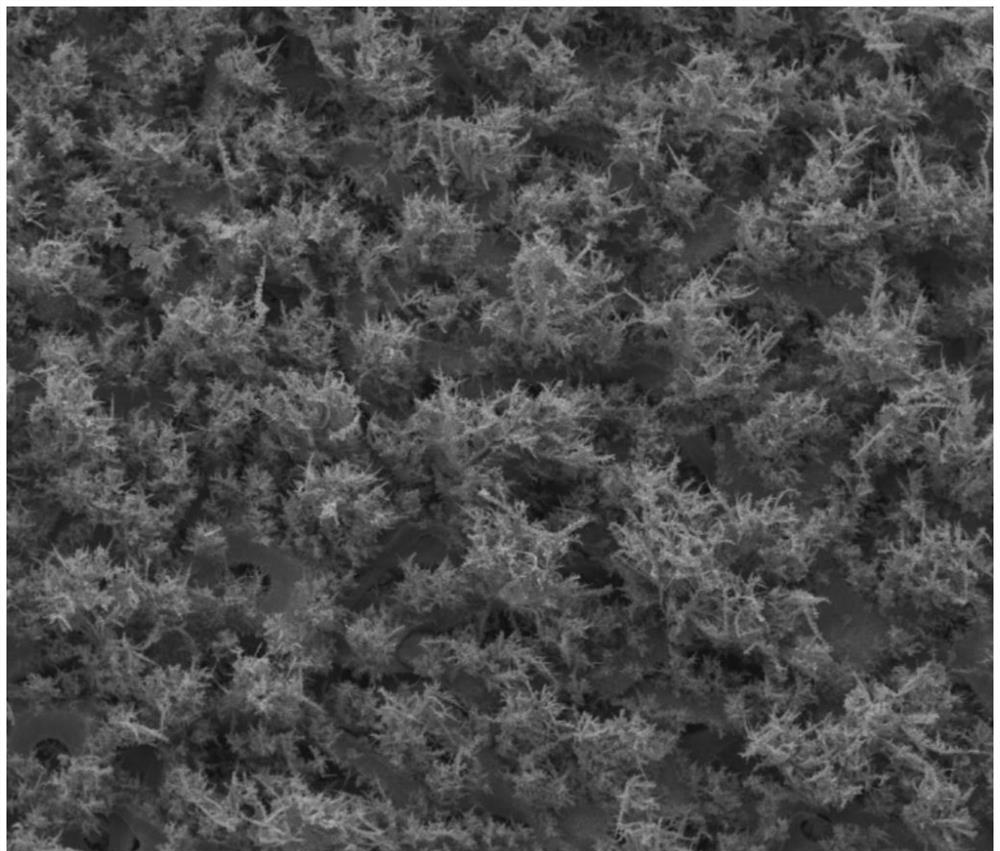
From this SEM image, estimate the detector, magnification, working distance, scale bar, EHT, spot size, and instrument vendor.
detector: ETD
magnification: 0.092 K X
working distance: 11.4 mm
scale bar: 1000000 nm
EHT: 20 kV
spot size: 3.5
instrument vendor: FEI